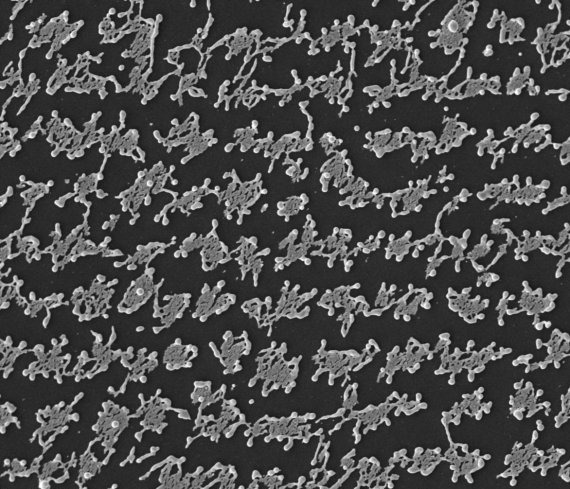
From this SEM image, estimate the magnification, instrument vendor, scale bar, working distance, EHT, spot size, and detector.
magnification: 10 K X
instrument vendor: FEI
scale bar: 10000 nm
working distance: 9.3 mm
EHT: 10 kV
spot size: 2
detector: ETD